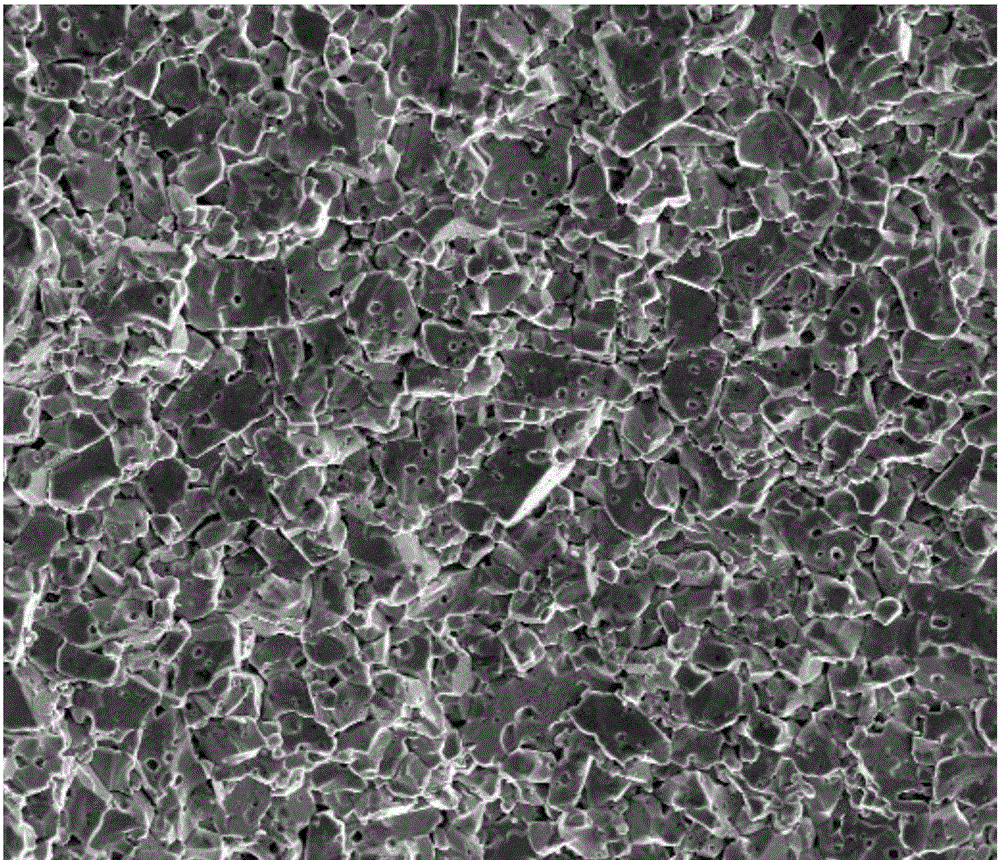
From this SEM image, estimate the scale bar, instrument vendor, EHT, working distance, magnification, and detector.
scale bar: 50000 nm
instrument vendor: FEI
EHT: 20 kV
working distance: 6.9 mm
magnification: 1 K X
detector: SE